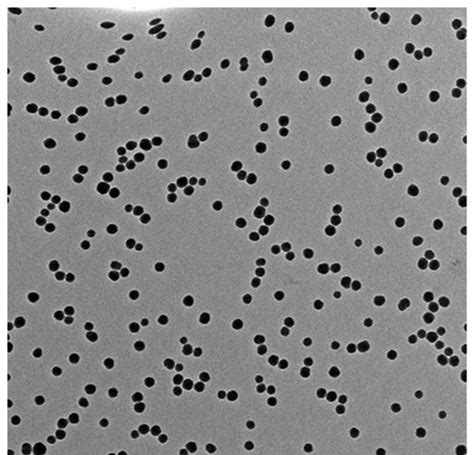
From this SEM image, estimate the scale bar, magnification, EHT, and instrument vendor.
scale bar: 500 nm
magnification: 17.5 K X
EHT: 120 kV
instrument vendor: other